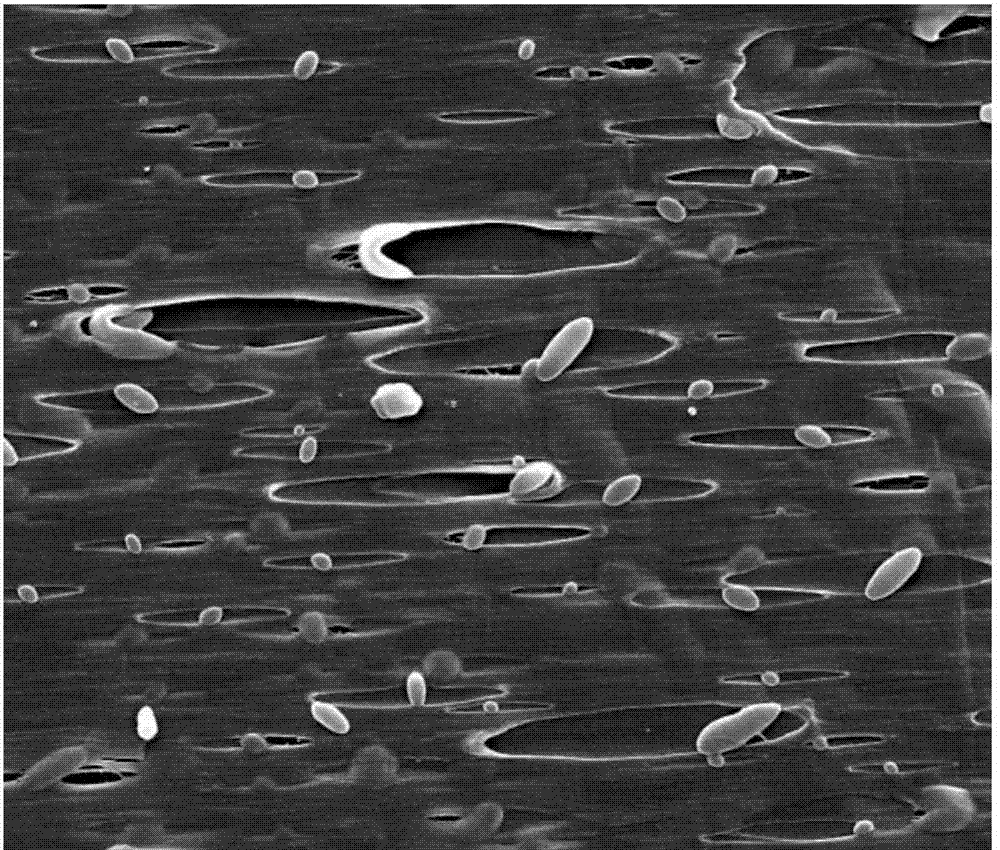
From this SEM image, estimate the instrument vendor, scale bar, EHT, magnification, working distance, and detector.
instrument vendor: FEI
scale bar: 10000 nm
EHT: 10 kV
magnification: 10 K X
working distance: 13.6 mm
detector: SE